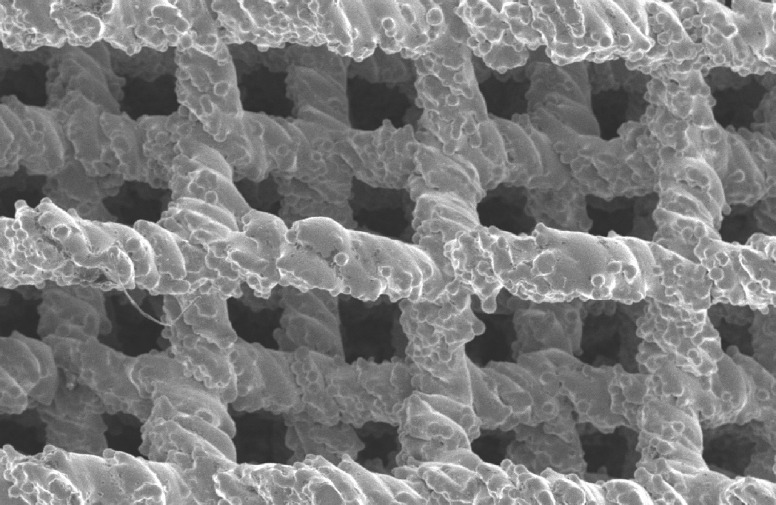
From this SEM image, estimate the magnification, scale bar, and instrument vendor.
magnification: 0.025 K X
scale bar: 1000000 nm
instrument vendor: Hitachi